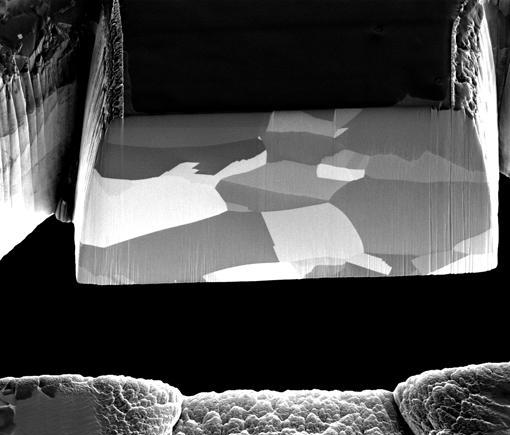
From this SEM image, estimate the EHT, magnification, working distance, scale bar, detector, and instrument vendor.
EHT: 30 kV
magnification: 3.25 K X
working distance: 19 mm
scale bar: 20000 nm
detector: ETD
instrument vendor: FEI